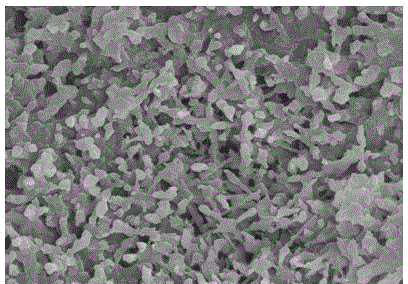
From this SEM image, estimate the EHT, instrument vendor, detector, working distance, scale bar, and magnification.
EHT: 15 kV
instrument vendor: Hitachi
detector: SE(U)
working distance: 9 mm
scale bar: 5000 nm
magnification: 10 K X